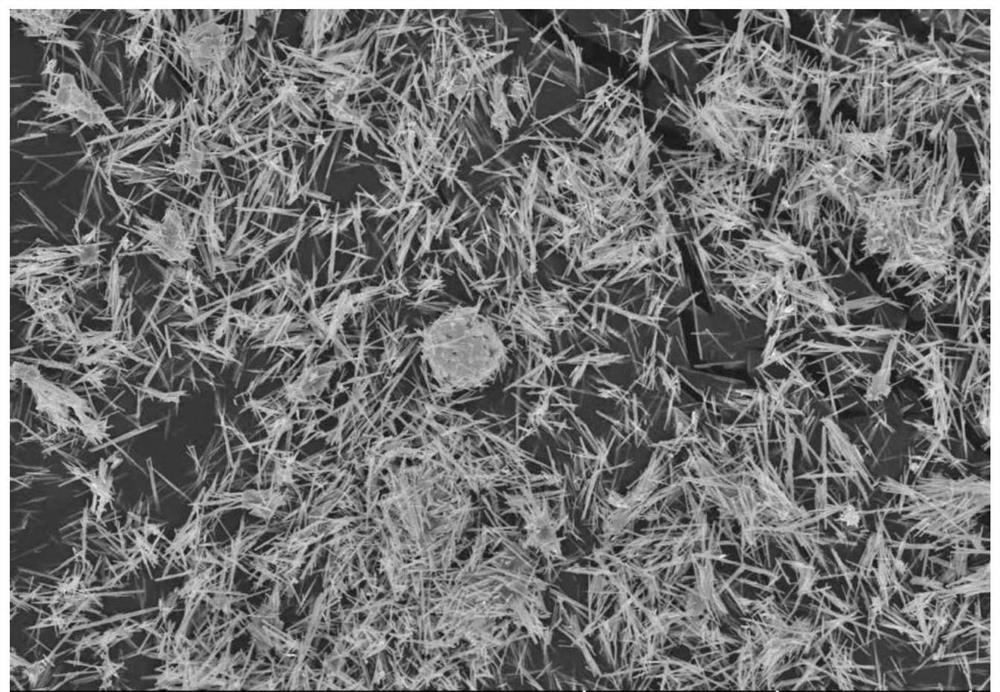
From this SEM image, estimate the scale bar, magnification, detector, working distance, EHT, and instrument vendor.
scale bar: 50000 nm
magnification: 1 K X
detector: SE(U)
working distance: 10.6 mm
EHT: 15 kV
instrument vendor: Hitachi